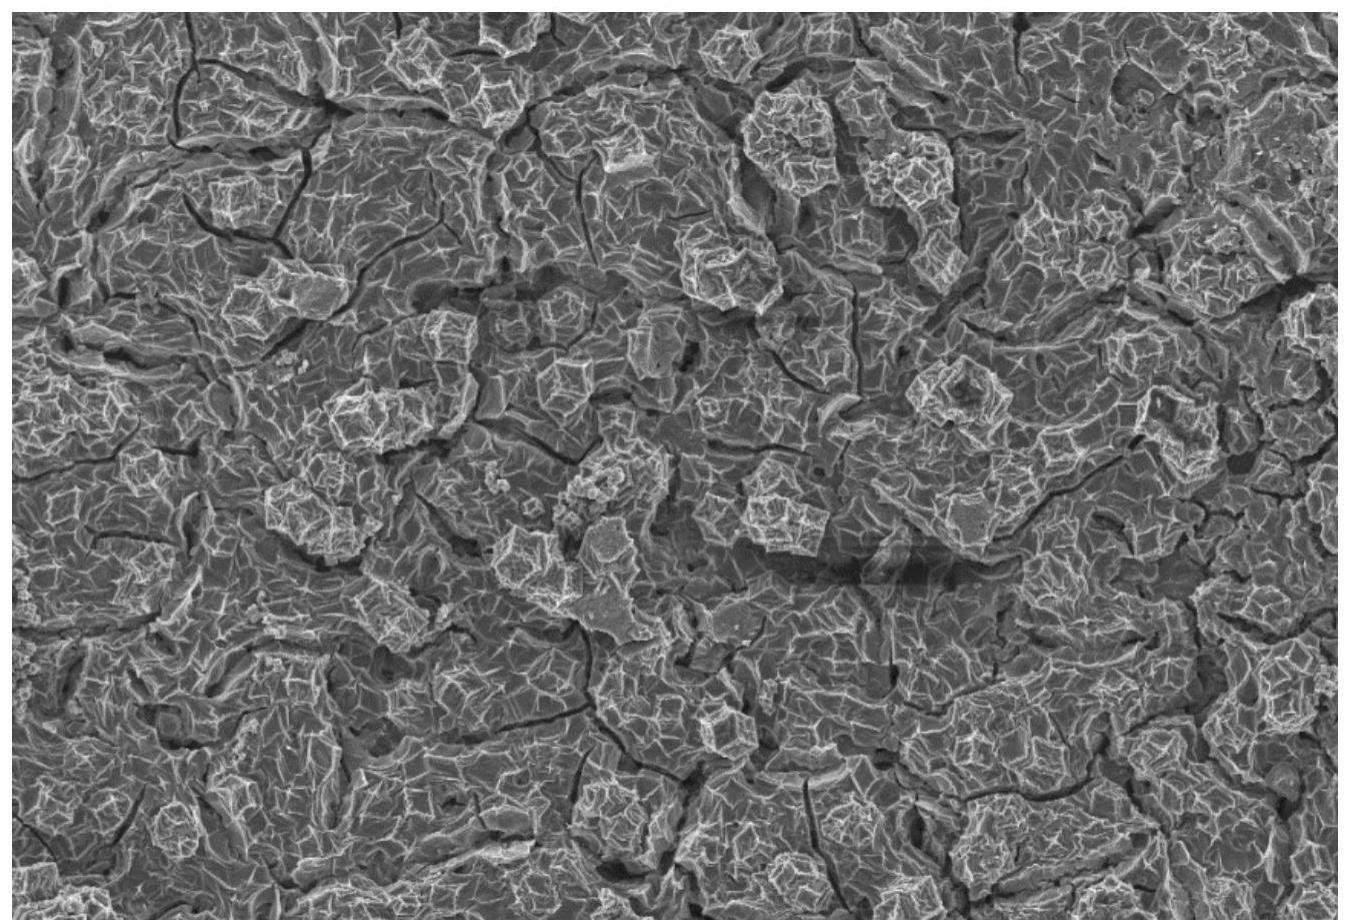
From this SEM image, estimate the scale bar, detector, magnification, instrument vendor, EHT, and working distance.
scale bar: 10000 nm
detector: InLens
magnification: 1 K X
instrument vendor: Zeiss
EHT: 5 kV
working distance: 4.3 mm